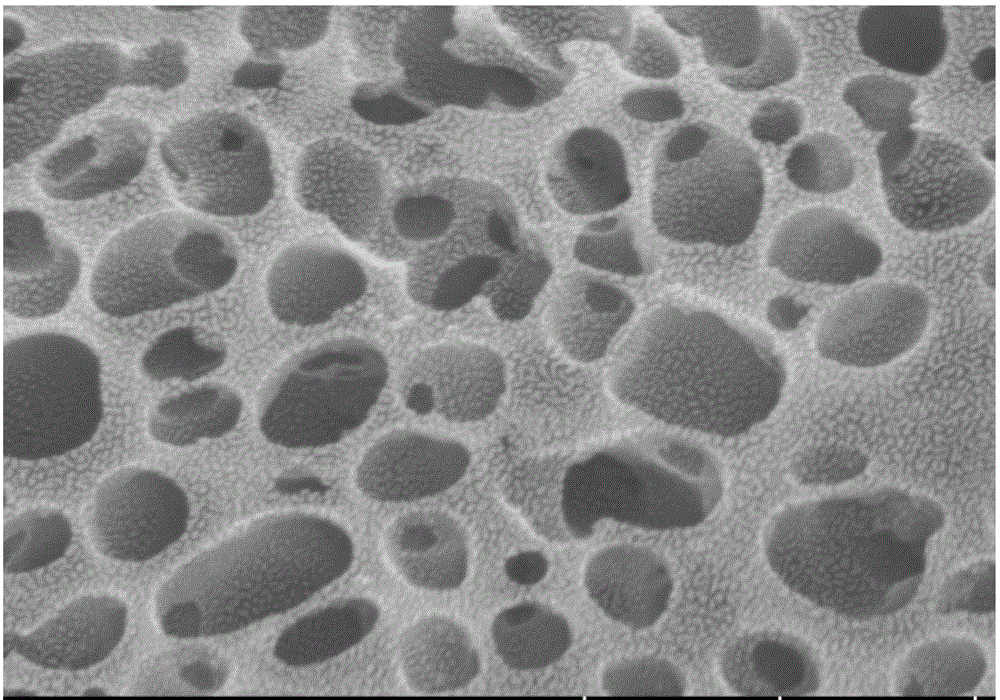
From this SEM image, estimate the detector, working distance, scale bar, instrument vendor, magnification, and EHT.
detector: SE(UL)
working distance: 8.5 mm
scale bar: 1000 nm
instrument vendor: Hitachi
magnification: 50 K X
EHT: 5 kV